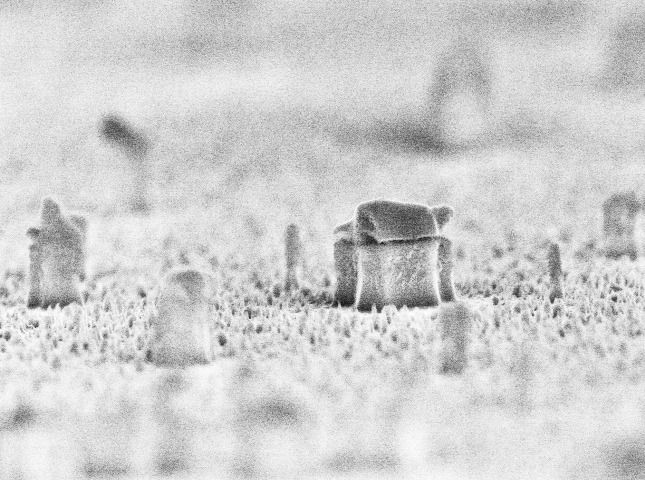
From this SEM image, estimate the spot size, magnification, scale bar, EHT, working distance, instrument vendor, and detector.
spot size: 2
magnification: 24.008 K X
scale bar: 1000 nm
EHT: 10 kV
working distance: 5.4 mm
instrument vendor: FEI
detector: TLD